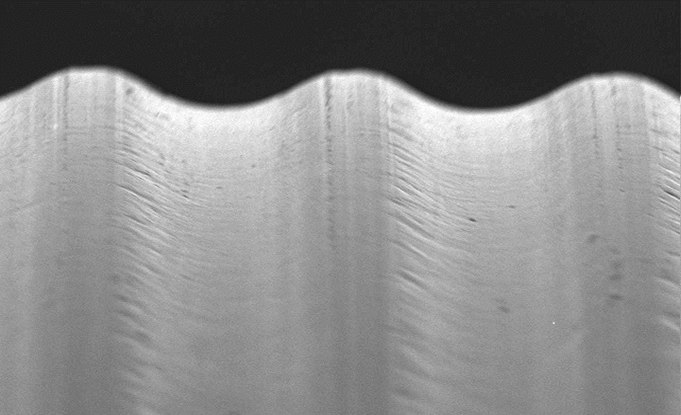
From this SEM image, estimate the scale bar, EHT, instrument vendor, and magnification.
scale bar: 50000 nm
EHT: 10 kV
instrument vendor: JEOL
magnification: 0.5 K X